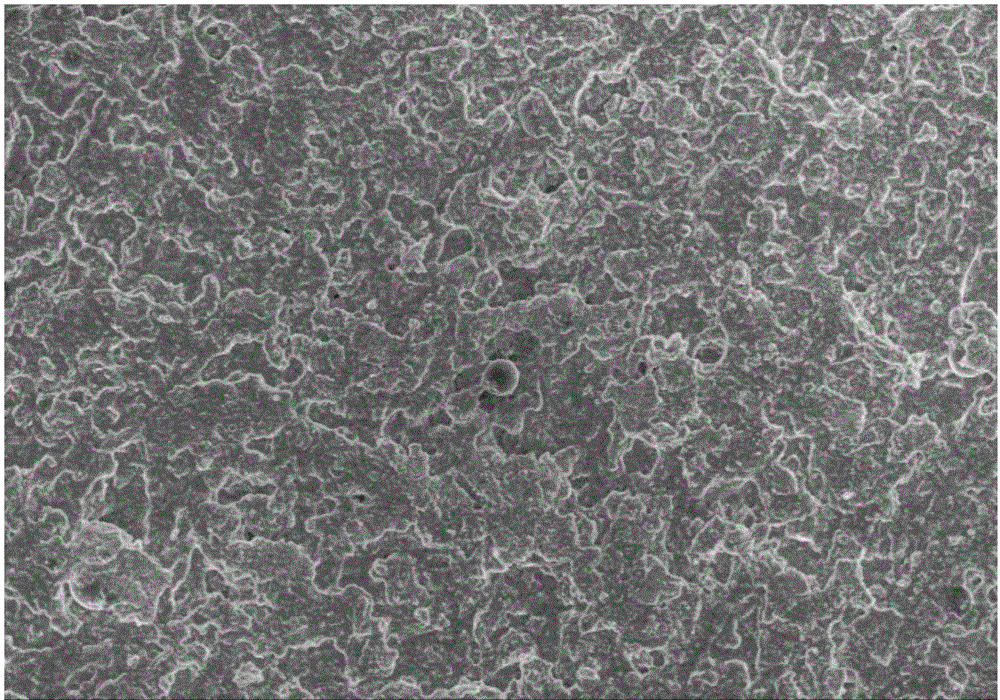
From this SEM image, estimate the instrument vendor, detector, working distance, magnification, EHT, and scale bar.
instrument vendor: Hitachi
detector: SE(M)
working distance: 8.7 mm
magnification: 0.5 K X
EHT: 15 kV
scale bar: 100000 nm